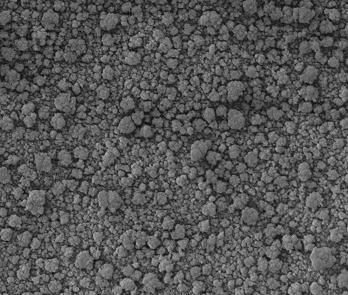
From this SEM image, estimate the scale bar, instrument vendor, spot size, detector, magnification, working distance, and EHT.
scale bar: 4000 nm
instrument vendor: FEI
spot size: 3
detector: ETD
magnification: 24 K X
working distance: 5.1 mm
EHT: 5 kV